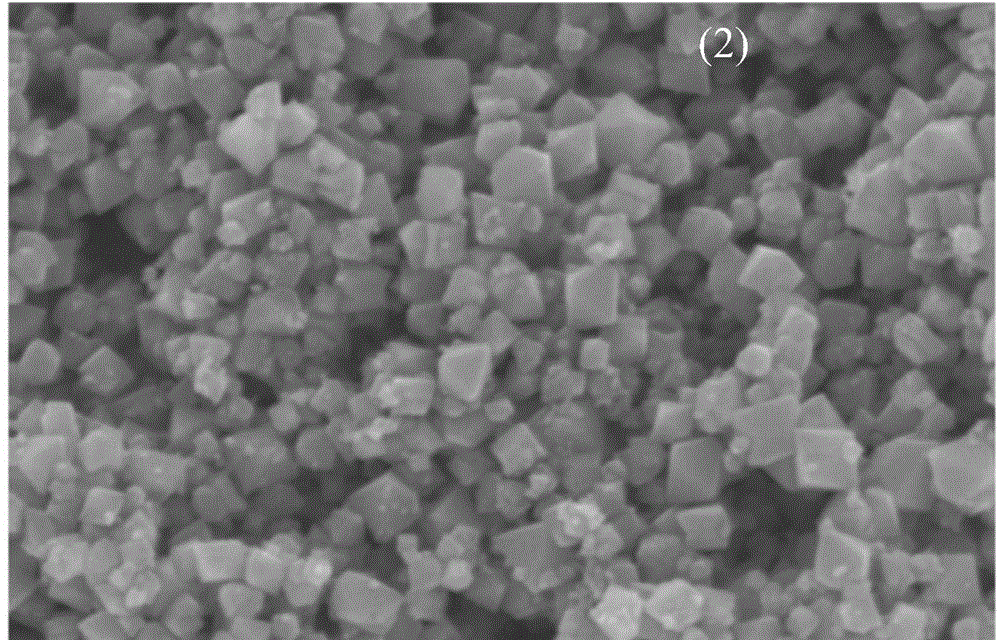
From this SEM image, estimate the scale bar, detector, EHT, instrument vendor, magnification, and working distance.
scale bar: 500 nm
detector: SE(M)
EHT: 5 kV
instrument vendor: Hitachi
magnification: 80 K X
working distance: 7.2 mm